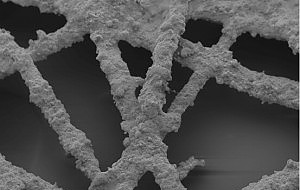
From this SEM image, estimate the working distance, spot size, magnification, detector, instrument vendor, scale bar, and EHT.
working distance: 10 mm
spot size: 3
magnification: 0.65 K X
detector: SE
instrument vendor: FEI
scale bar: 100000 nm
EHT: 5 kV